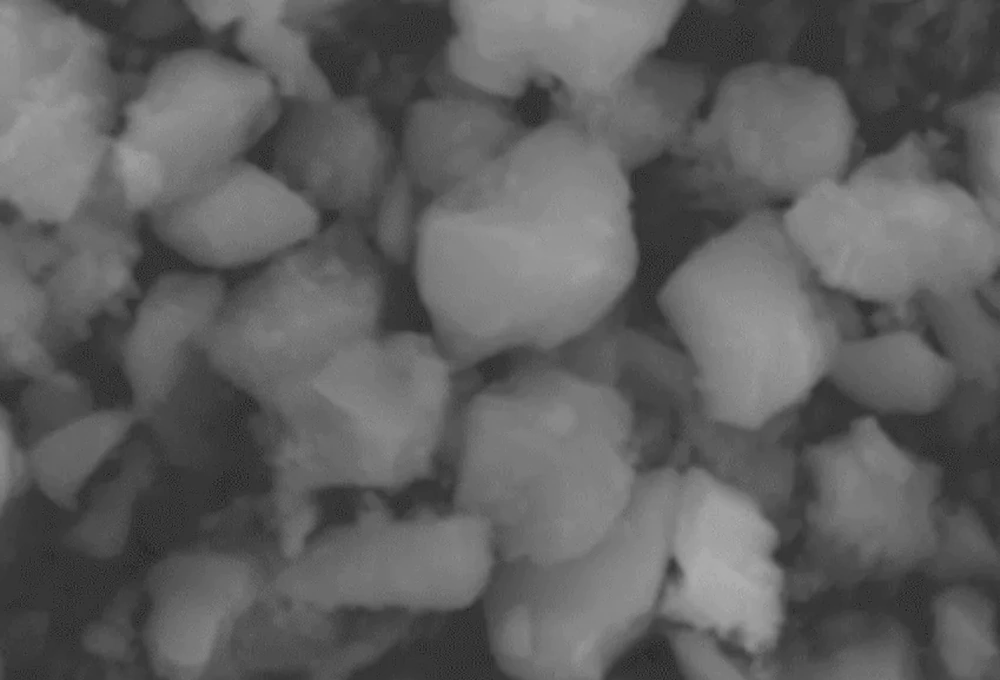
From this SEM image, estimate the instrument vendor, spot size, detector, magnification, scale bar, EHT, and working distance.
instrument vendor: JEOL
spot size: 52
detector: SEI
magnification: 10 K X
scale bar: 1000 nm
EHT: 20 kV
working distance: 10 mm